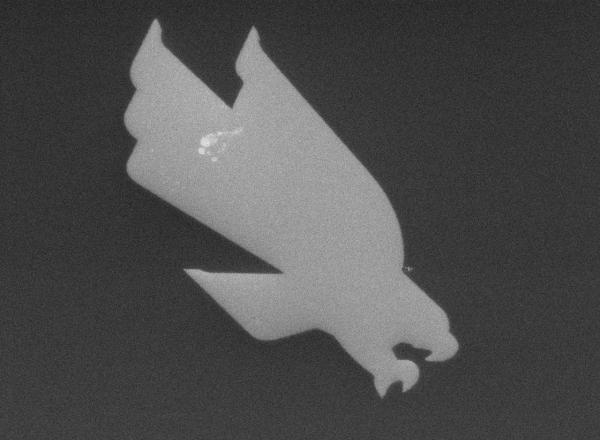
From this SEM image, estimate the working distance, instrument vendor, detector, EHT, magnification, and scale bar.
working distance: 10.2 mm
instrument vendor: JEOL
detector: SEI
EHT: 15 kV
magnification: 0.6 K X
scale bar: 10000 nm